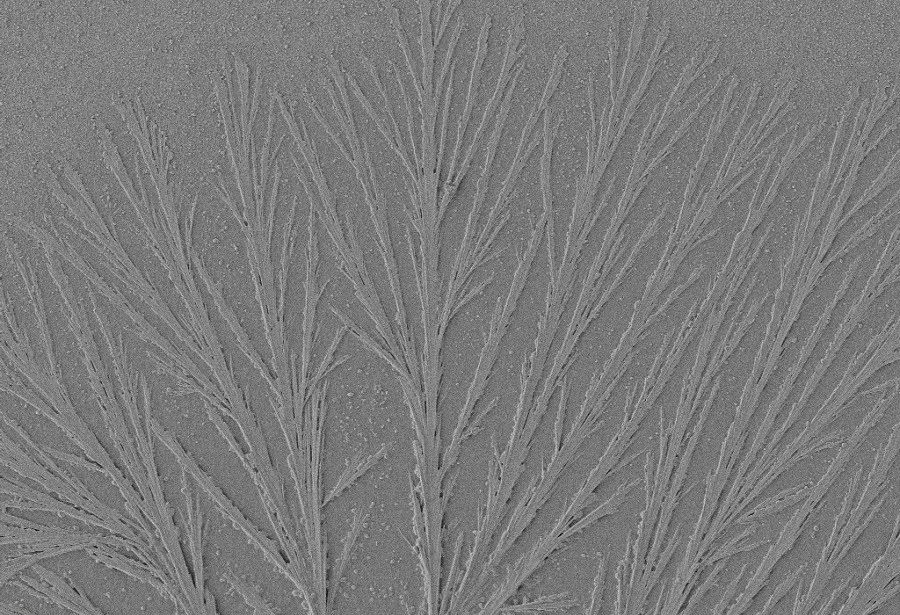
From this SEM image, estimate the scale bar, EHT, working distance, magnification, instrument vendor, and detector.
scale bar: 20000 nm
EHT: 3 kV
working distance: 8.1 mm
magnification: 0.5 K X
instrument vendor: Zeiss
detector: SE2